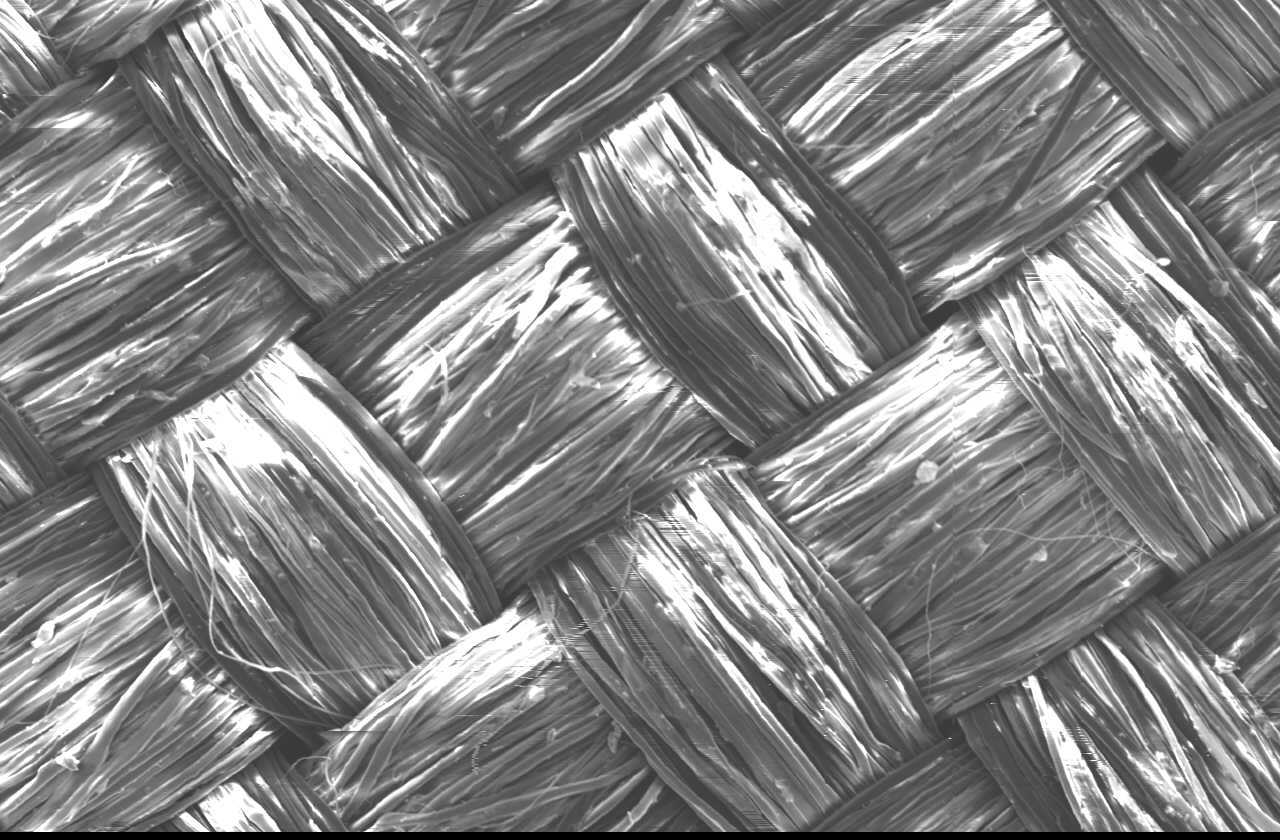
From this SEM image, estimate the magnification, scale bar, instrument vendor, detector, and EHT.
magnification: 0.085 K X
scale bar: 200000 nm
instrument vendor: JEOL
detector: SEI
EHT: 15 kV